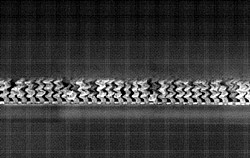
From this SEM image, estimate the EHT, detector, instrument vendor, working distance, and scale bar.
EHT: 2 kV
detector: InLens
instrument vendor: Zeiss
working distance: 3 mm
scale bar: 200 nm